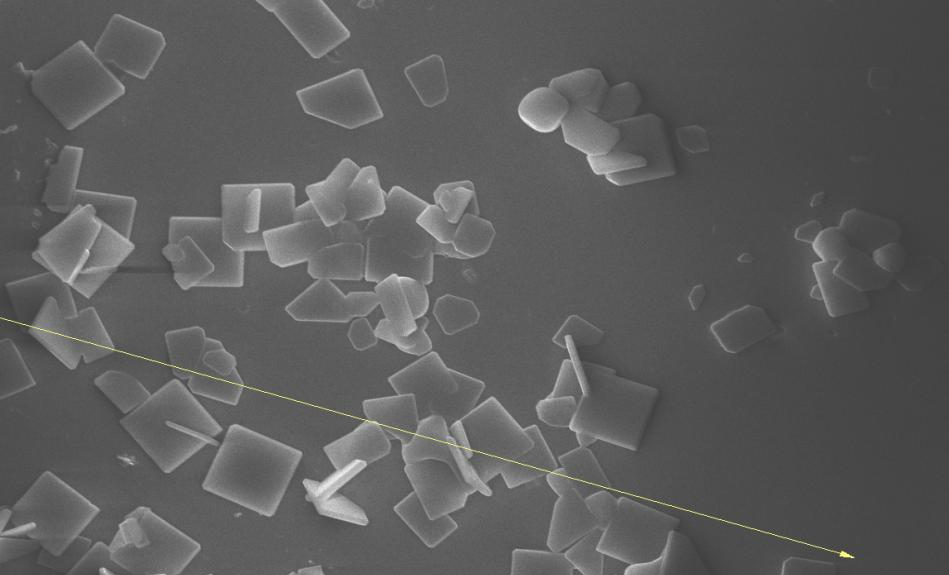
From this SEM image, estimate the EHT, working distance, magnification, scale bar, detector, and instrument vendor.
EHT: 15 kV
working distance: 3.9 mm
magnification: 2.419 K X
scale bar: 20000 nm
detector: SE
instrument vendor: other